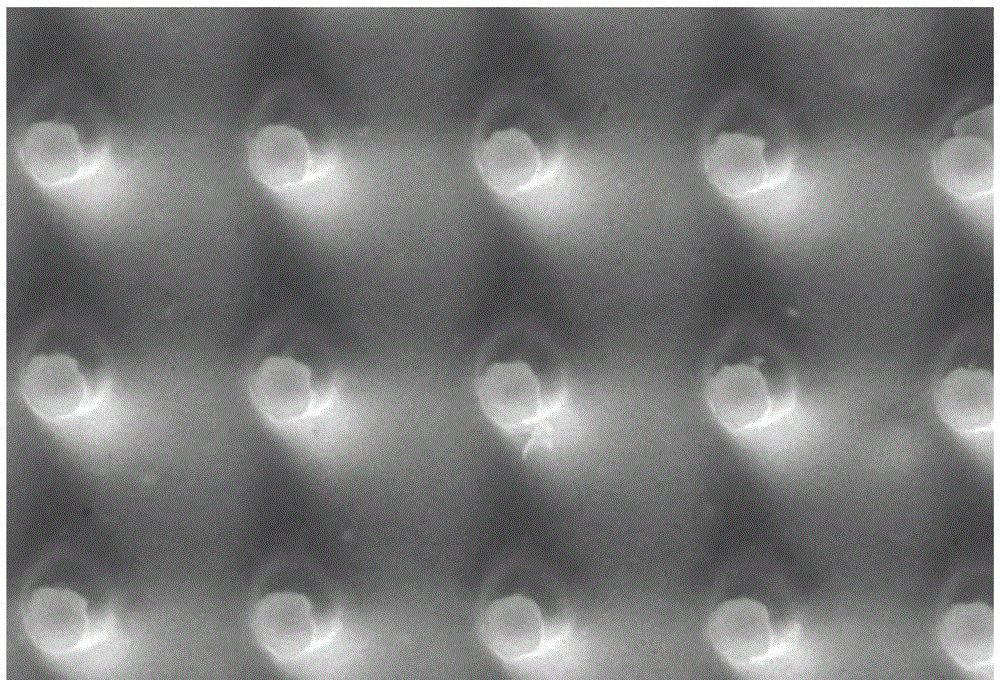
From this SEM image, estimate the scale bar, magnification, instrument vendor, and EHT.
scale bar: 5000 nm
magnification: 3 K X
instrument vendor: JEOL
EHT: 20 kV